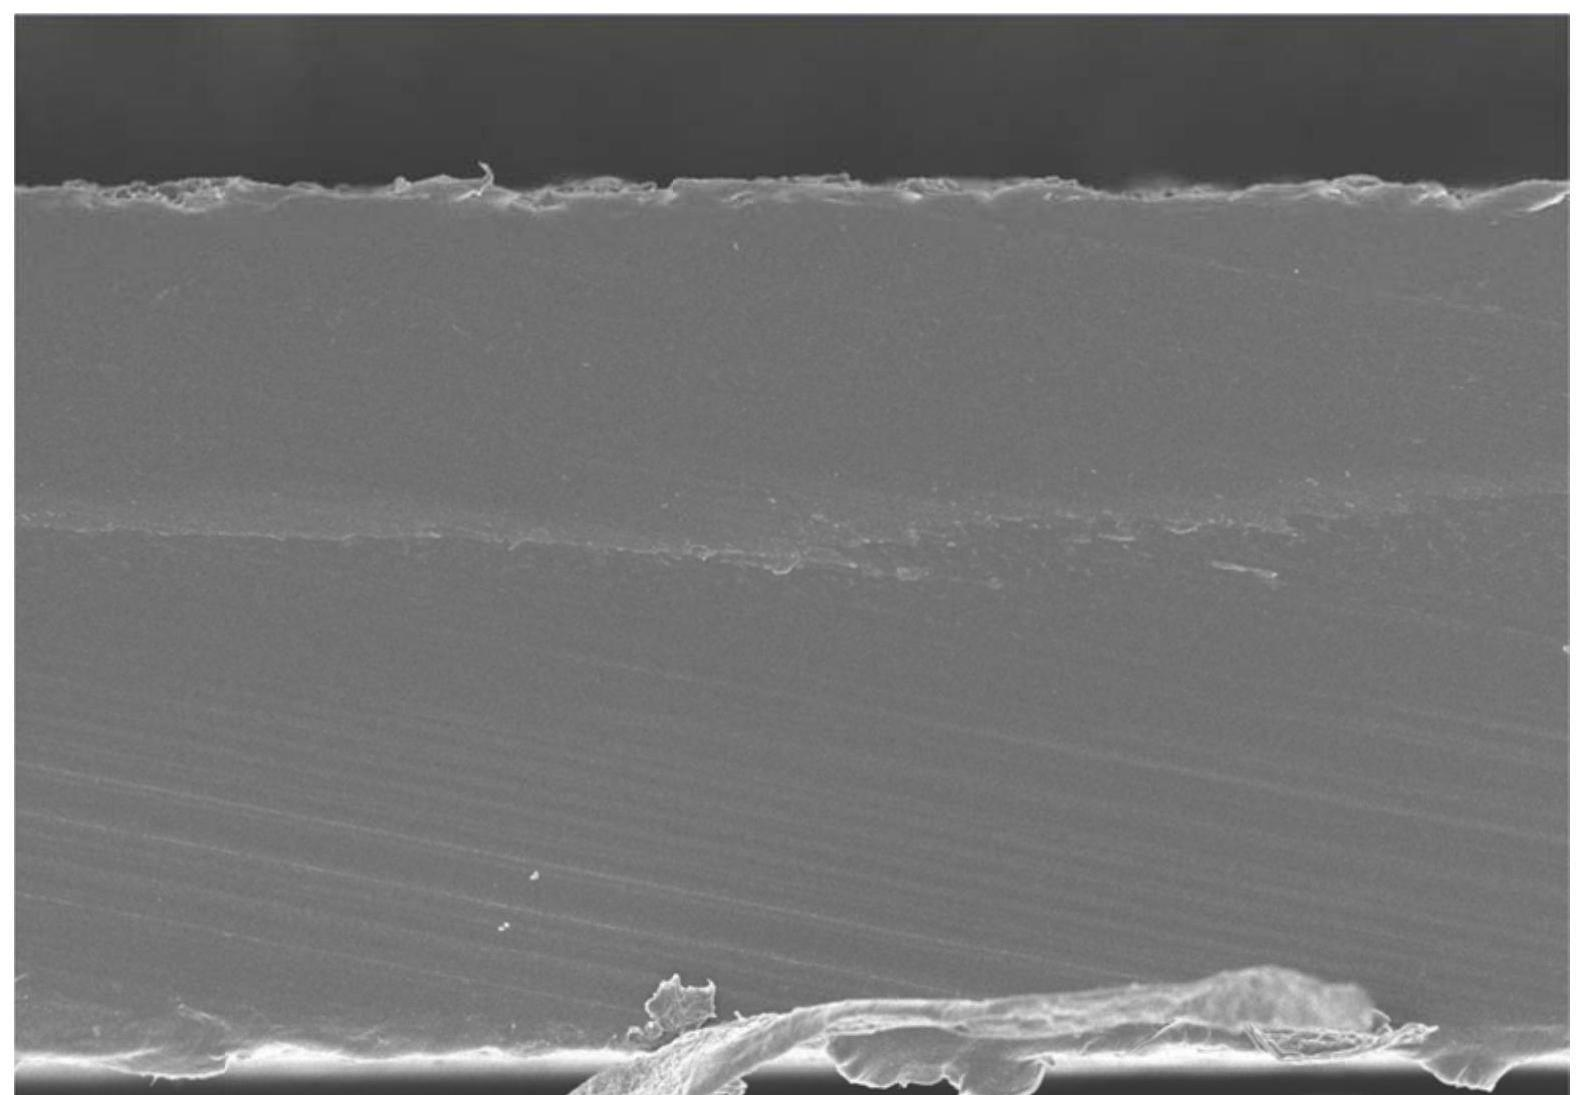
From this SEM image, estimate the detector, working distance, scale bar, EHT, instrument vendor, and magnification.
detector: SE(UL)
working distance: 6.3 mm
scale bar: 50000 nm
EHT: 5 kV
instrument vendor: Hitachi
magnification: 0.6 K X